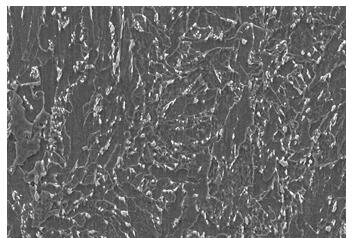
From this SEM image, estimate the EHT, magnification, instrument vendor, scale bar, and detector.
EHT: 20 kV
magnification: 2 K X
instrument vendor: JEOL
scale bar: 10000 nm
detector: SEI